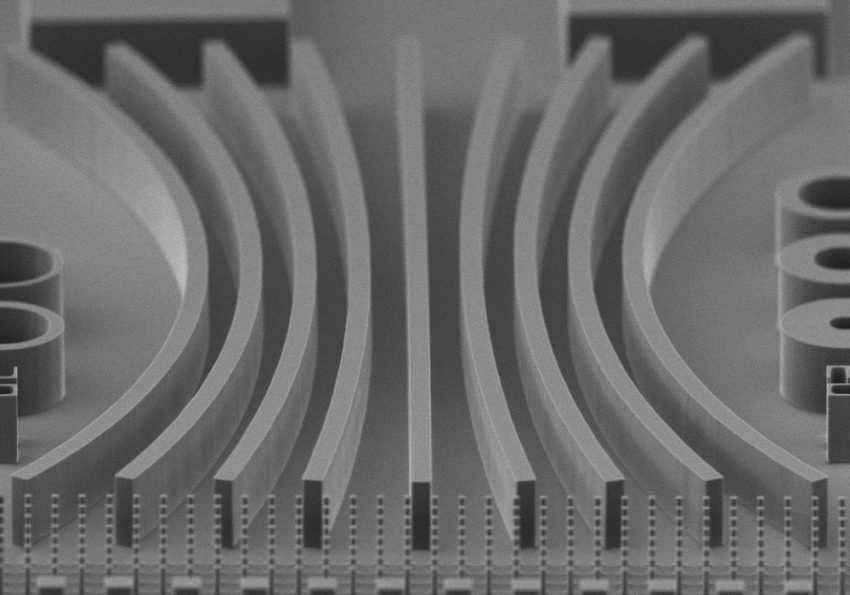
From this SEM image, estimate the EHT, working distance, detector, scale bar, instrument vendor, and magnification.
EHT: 5 kV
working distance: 9.5 mm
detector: SE(M)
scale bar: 50000 nm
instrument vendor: Hitachi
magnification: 1 K X